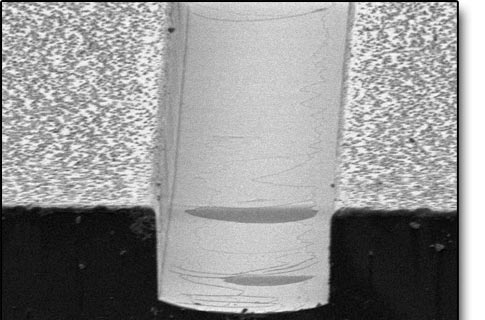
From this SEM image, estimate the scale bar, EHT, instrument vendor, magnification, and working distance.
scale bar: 33300 nm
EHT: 5 kV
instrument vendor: Hitachi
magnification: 0.901 K X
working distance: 15 mm